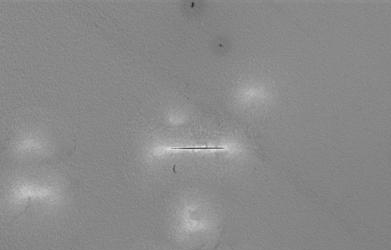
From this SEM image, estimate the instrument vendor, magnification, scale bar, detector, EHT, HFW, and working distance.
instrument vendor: FEI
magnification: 2.5 K X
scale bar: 50000 nm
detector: ETD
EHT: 5 kV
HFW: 166 µm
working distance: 7.8353 mm